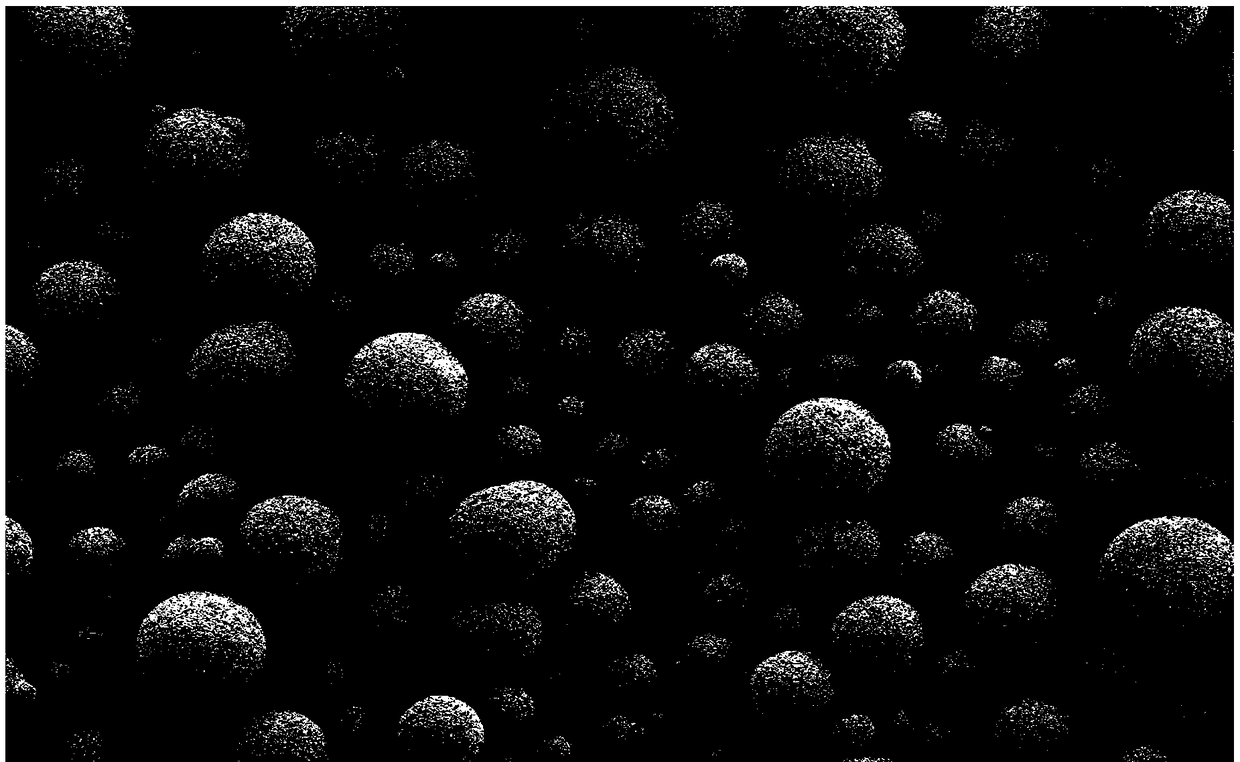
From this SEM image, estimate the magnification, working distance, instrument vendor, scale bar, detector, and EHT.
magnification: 0.3 K X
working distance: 4.7 mm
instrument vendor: Zeiss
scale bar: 20000 nm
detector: SE2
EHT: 5 kV